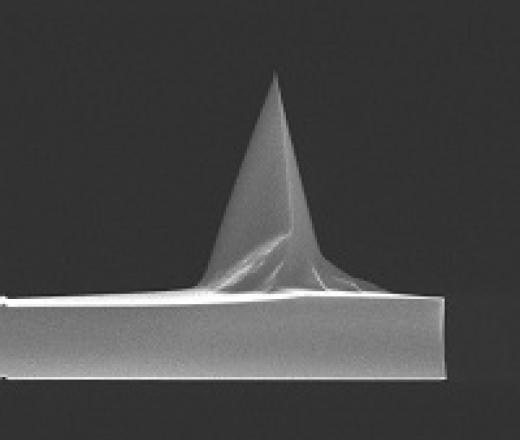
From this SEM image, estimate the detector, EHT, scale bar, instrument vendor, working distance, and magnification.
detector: TLD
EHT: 15 kV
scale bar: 10000 nm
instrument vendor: FEI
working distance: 5.5 mm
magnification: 3.5 K X